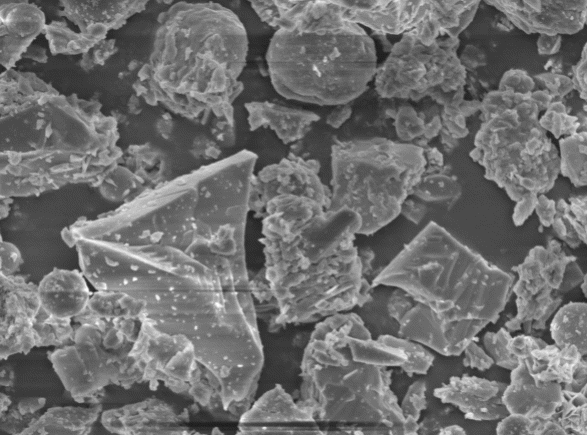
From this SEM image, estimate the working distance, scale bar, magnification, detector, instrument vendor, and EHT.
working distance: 16.8 mm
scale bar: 1000 nm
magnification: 5 K X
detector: SEI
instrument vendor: JEOL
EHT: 5 kV